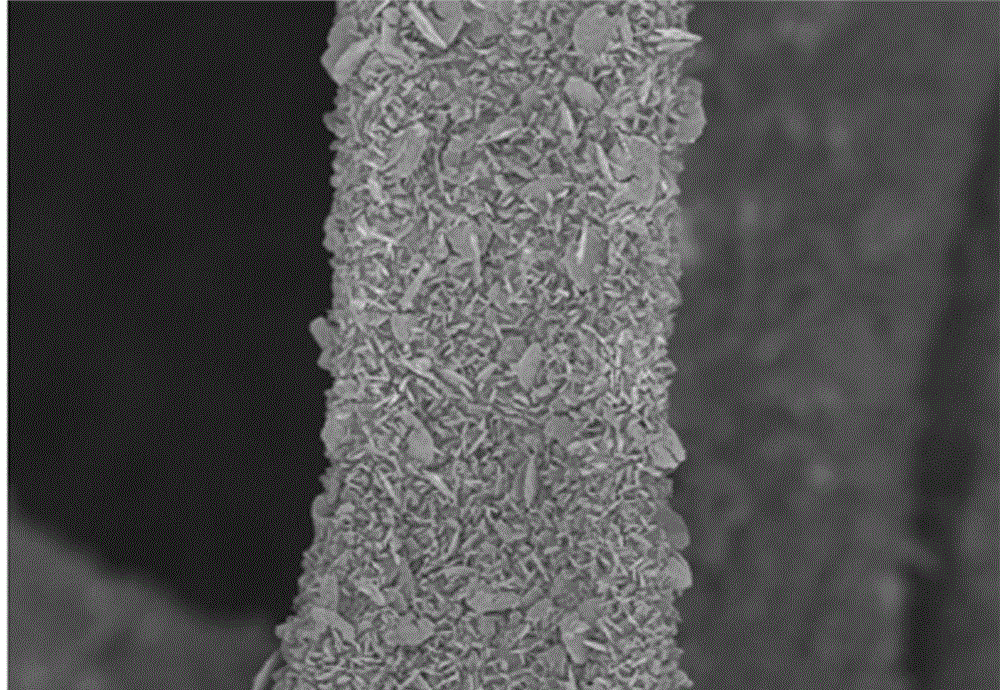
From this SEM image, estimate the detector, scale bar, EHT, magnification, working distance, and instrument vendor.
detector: SE(M)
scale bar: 50000 nm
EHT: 3 kV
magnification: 0.7 K X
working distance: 4.8 mm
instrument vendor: Hitachi